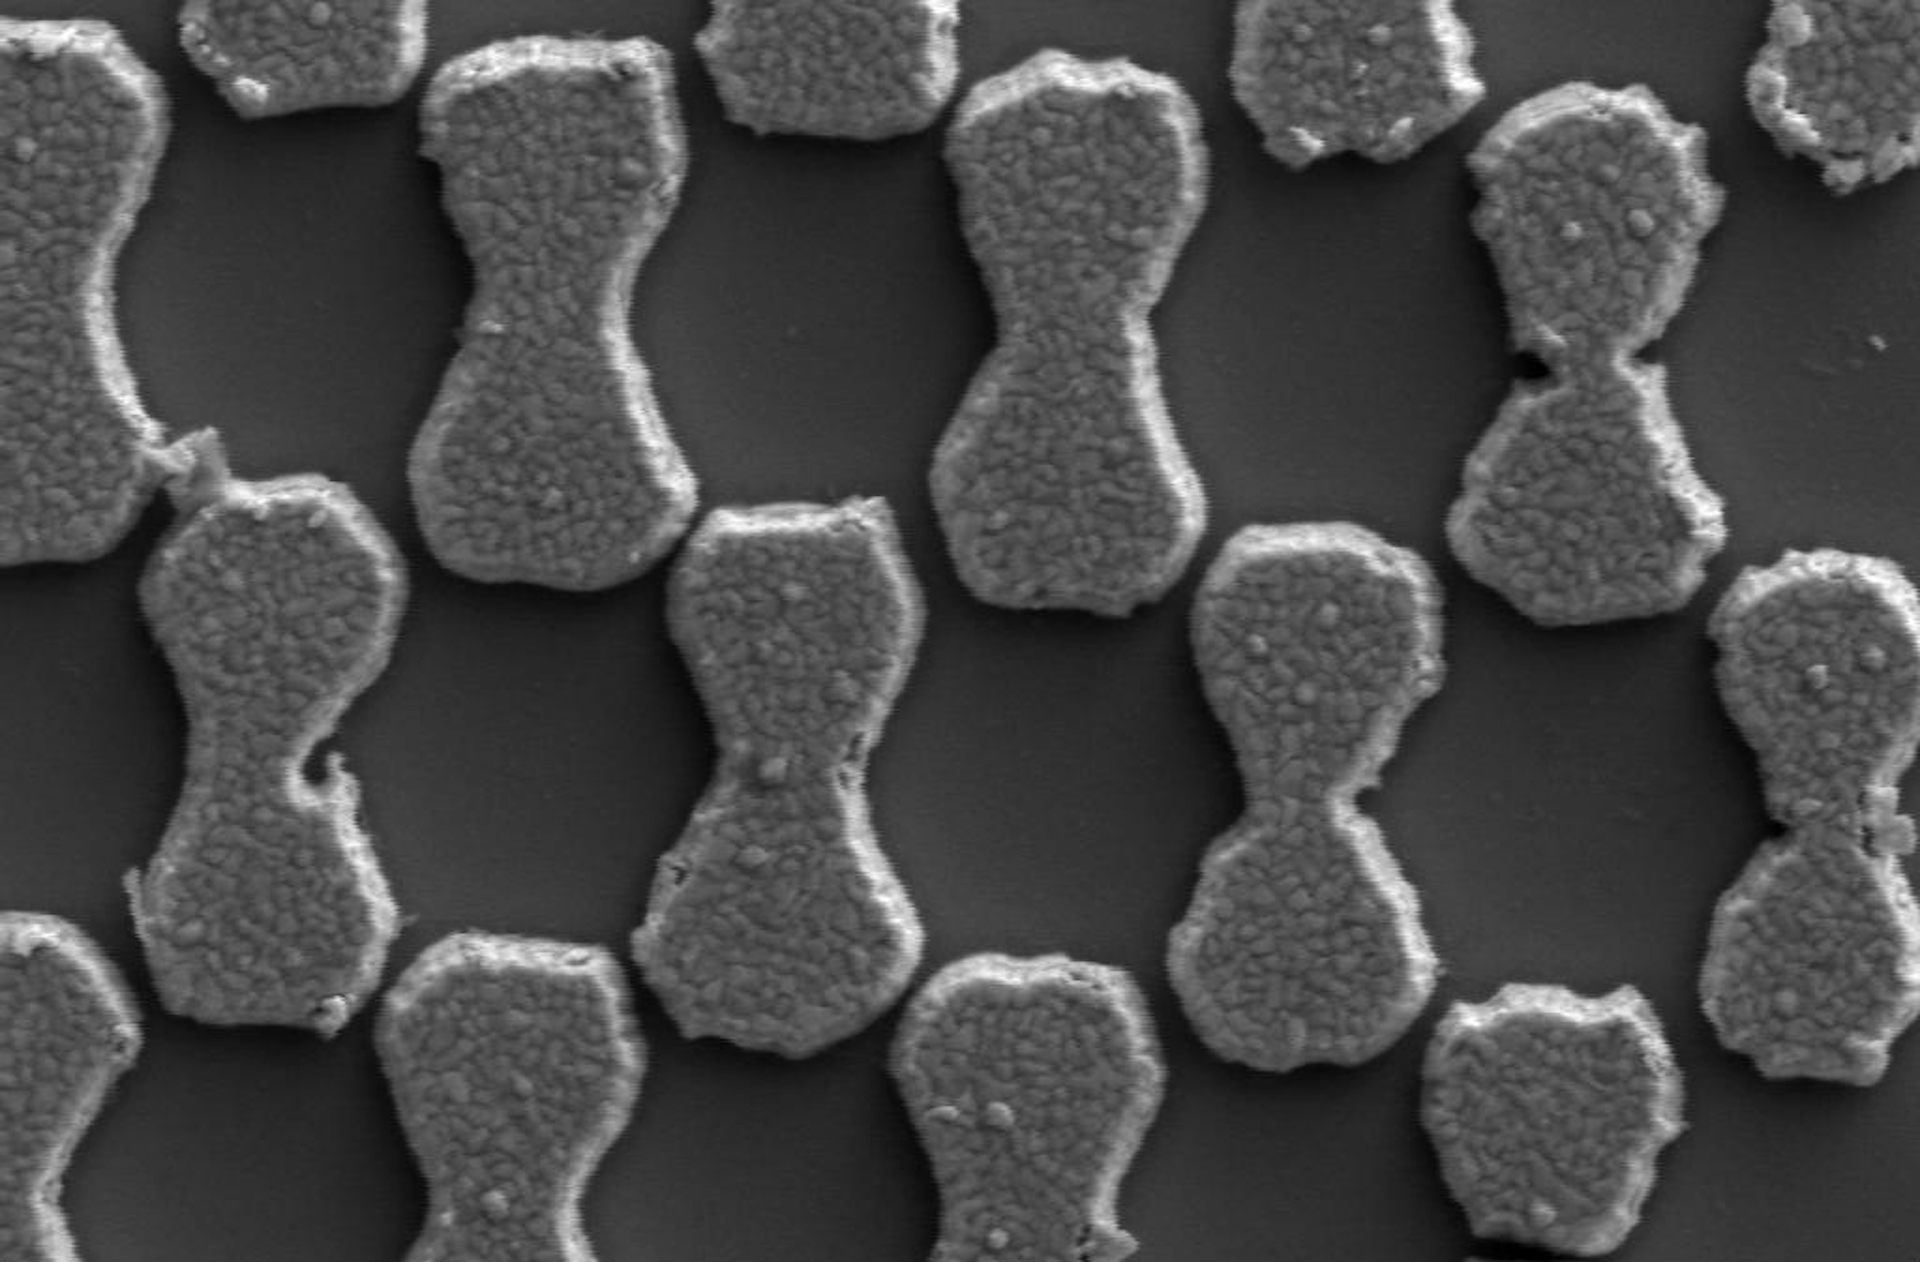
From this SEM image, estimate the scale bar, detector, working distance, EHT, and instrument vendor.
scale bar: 1000 nm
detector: SE2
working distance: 27.9 mm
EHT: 5 kV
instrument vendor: Zeiss